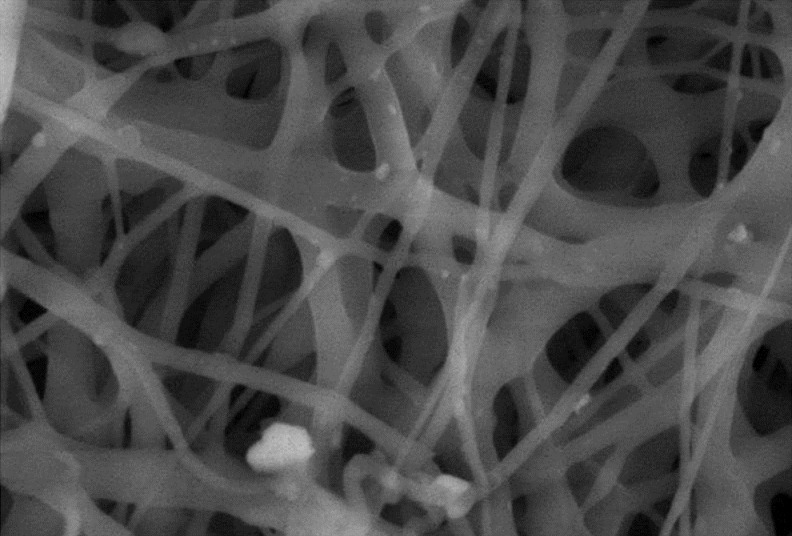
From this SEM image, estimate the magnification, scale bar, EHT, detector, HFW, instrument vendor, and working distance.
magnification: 5 K X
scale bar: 10000 nm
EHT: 15 kV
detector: LFD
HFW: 29.8 µm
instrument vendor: FEI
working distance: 9.7 mm